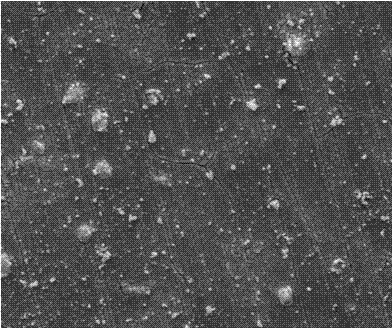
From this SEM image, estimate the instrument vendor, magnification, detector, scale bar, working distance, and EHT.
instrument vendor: FEI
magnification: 1 K X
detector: SE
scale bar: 50000 nm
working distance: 12.2 mm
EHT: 20 kV